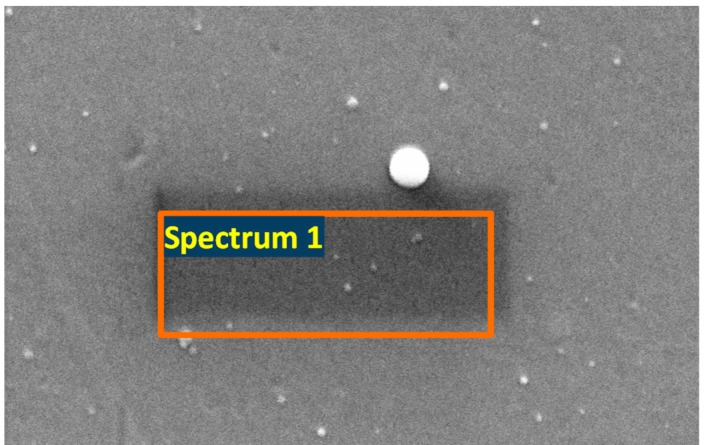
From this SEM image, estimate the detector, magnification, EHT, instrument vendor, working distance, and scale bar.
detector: SE1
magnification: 51.78 K X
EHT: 25 kV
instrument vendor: Zeiss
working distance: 6.5 mm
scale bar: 200 nm